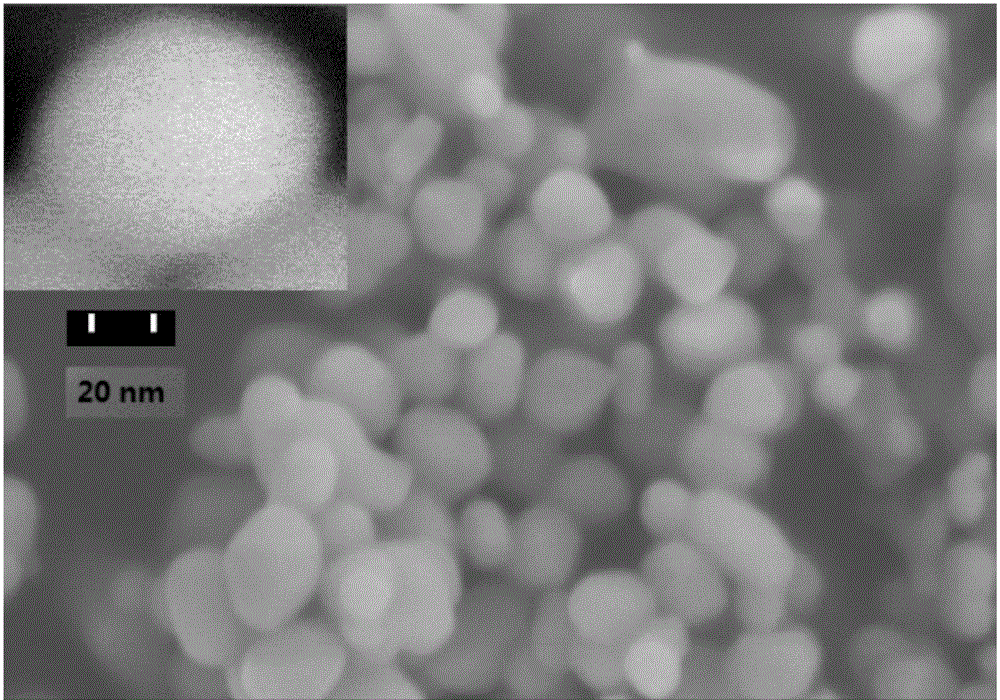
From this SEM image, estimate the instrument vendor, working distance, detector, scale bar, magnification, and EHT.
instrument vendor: Hitachi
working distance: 14.1 mm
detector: SE(M)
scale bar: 500 nm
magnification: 100 K X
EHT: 5 kV